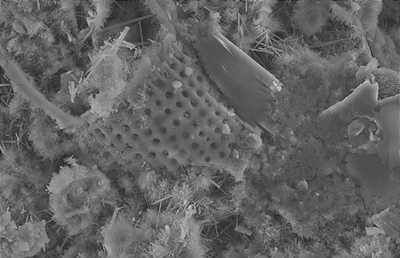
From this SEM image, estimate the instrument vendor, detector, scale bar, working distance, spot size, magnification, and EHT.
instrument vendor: FEI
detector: SE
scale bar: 5000 nm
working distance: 6 mm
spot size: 3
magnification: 10 K X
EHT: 10 kV